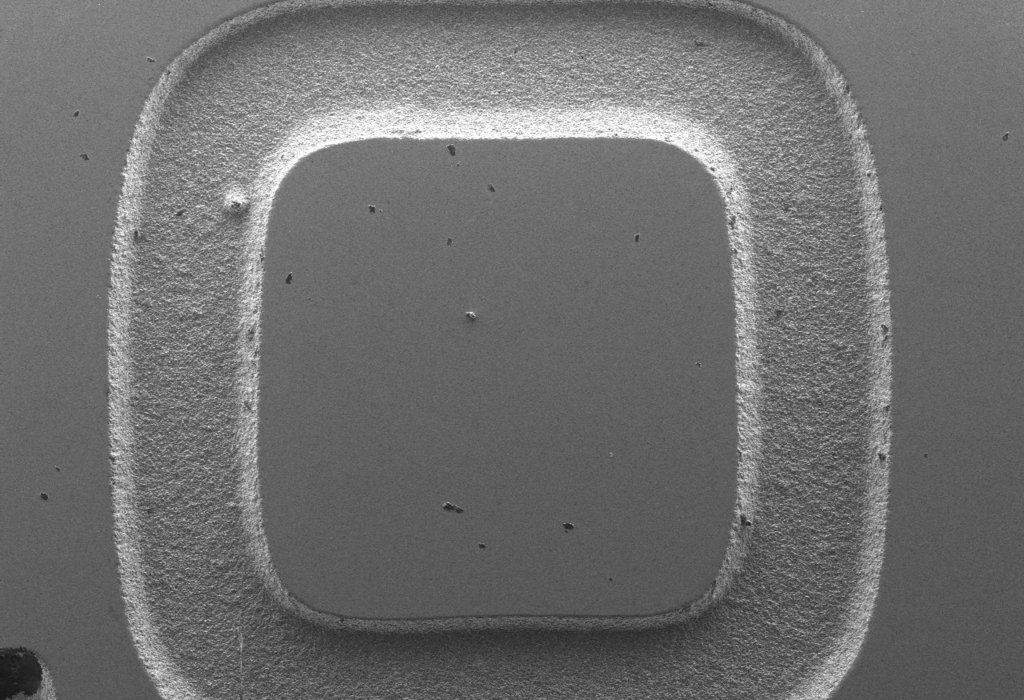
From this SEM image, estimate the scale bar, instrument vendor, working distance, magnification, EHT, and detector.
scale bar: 1000000 nm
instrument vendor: Hitachi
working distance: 5.9 mm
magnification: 0.035 K X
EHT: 10 kV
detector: LM(L)